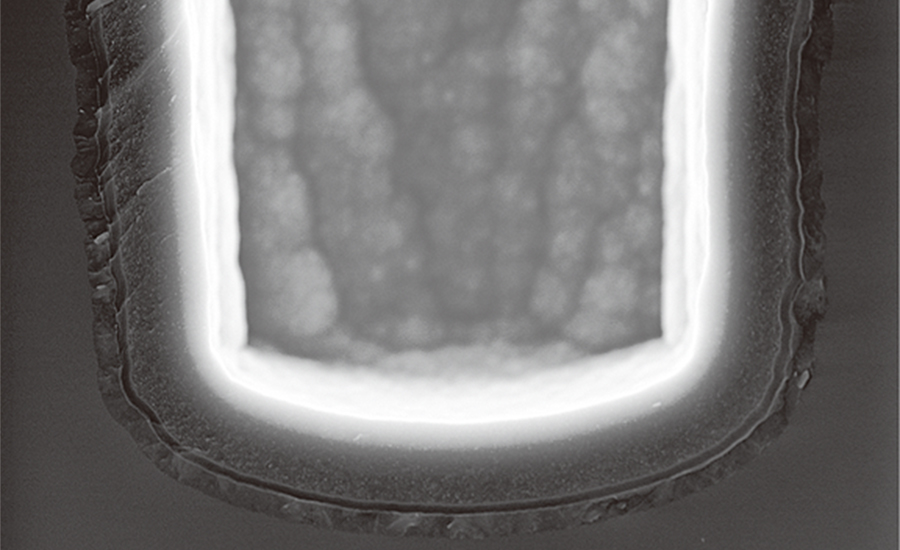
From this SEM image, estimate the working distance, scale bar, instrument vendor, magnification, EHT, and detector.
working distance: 2 mm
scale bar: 2000 nm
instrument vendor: Hitachi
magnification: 25 K X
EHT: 5 kV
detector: SE(U)